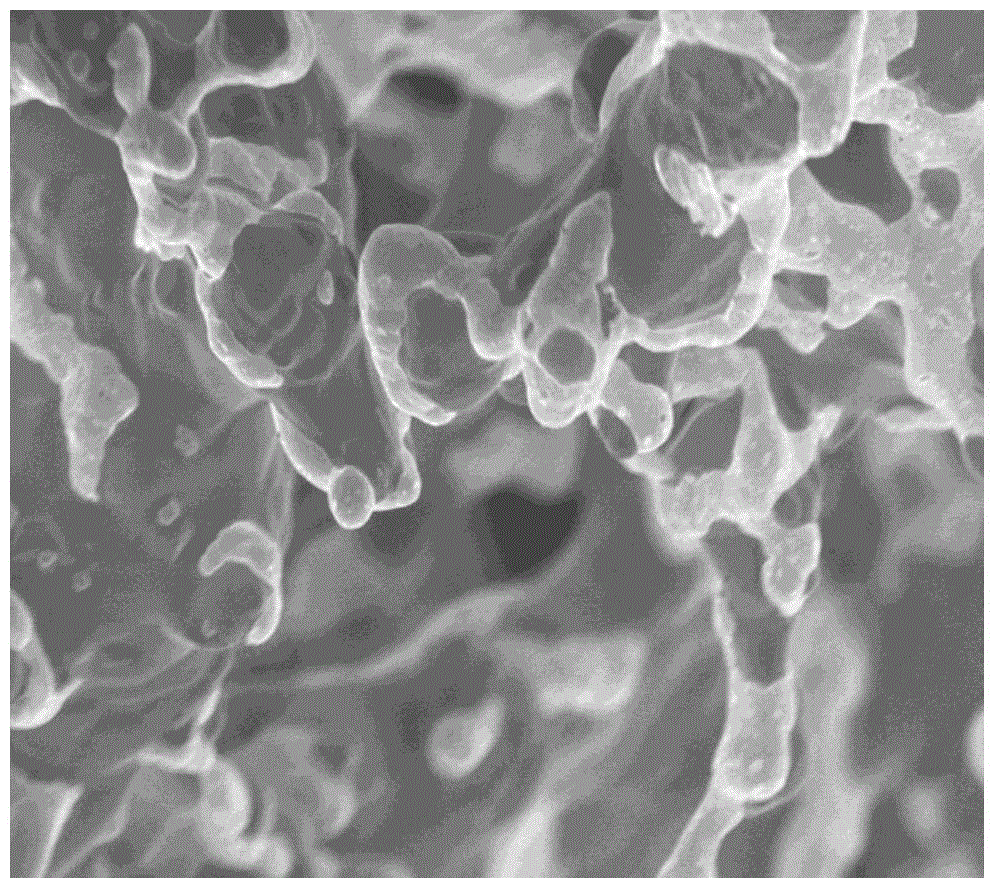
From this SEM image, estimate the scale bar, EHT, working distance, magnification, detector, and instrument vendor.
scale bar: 20000 nm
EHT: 1 kV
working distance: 2.5 mm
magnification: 2.52 K X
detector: SE+BSE(TU)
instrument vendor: Hitachi